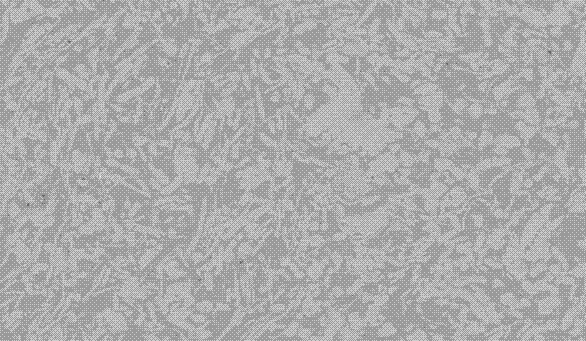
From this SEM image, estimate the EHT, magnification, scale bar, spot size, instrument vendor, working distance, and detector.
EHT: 15 kV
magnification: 0.8 K X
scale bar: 20000 nm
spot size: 5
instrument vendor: FEI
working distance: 21.7 mm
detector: BSE